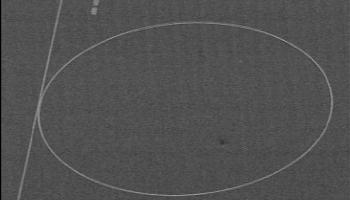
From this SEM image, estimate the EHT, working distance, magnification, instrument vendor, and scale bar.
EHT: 20 kV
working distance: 6 mm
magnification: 0.25 K X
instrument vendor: JEOL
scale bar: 100000 nm